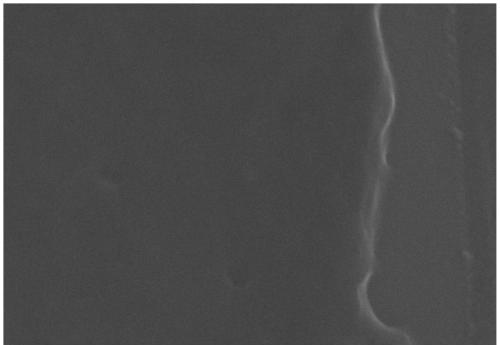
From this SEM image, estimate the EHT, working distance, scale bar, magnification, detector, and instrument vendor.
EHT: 15 kV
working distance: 11.5 mm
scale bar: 100 nm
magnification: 317.62 K X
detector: SE2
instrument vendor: Zeiss